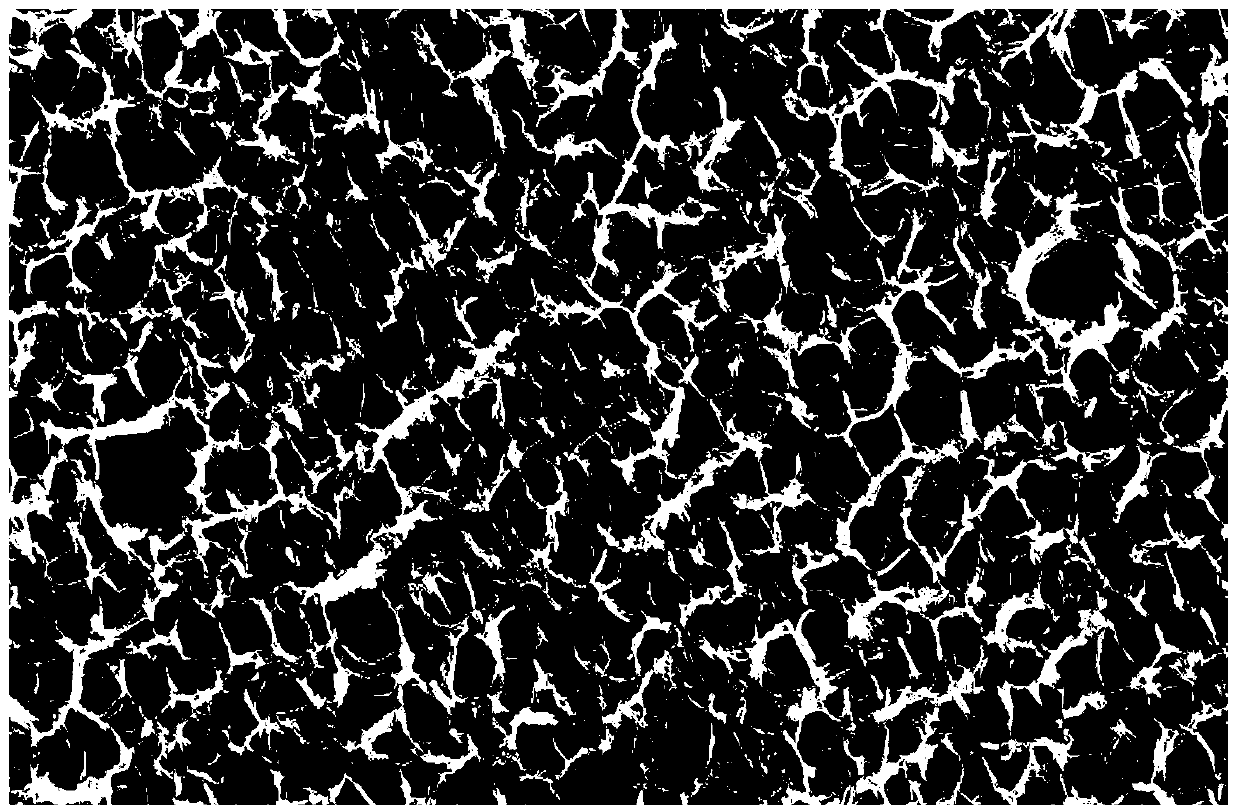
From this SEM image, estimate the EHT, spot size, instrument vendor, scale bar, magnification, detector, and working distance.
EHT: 15 kV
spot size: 3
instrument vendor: FEI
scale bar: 200000 nm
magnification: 0.1 K X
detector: SE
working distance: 20.1 mm